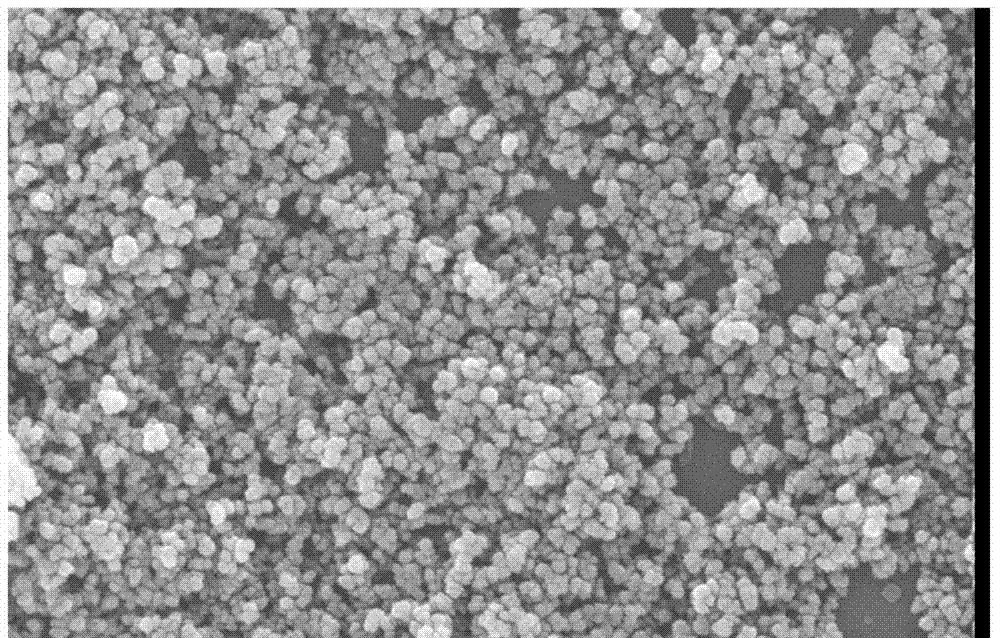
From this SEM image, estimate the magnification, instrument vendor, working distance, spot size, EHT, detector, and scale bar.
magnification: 50 K X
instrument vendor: FEI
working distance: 6.5 mm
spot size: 4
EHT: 20 kV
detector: TLD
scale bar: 1000 nm